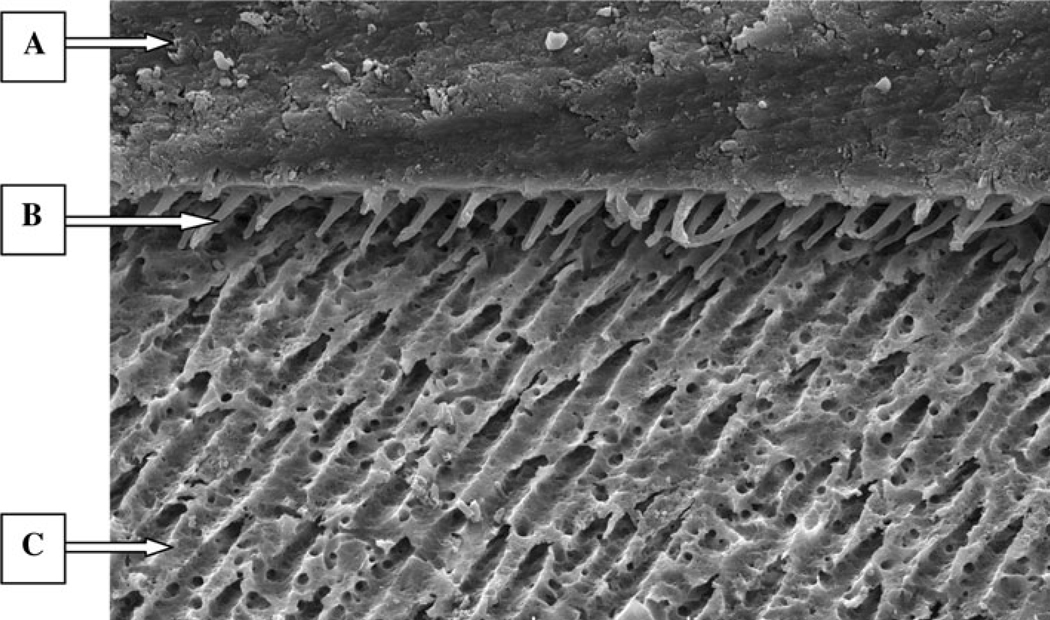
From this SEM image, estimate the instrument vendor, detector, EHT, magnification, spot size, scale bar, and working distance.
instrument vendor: FEI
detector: SE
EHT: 15 kV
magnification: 1 K X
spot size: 4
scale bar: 20000 nm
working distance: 17.6 mm